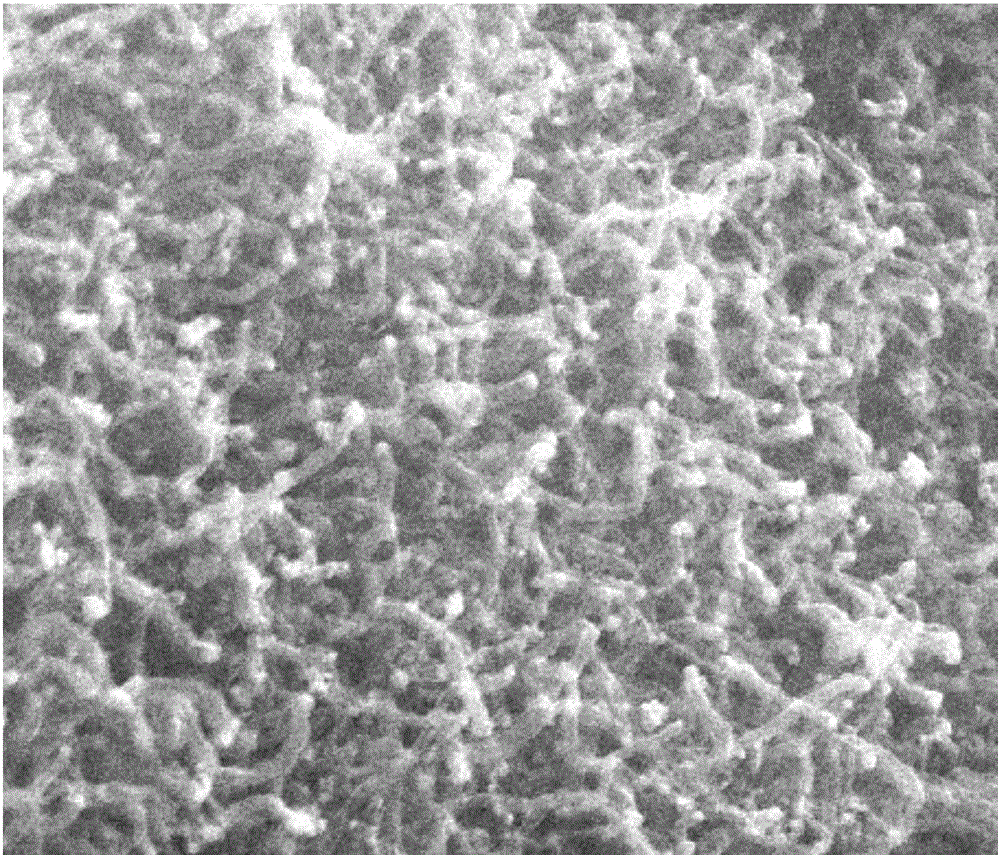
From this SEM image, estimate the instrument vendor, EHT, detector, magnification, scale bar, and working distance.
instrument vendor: FEI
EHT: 20 kV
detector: SE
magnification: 50 K X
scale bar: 1000 nm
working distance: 4.5 mm